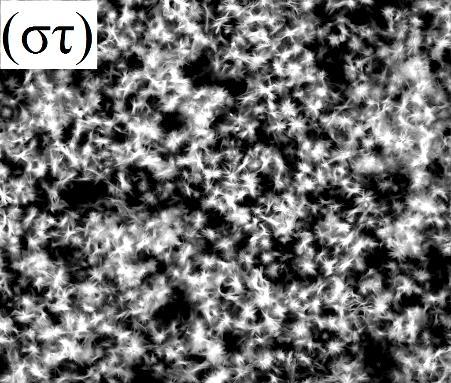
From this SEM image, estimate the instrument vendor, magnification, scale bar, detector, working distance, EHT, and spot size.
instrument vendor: FEI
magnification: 0.2 K X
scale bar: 300000 nm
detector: SSD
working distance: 9.7 mm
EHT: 25 kV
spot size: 5